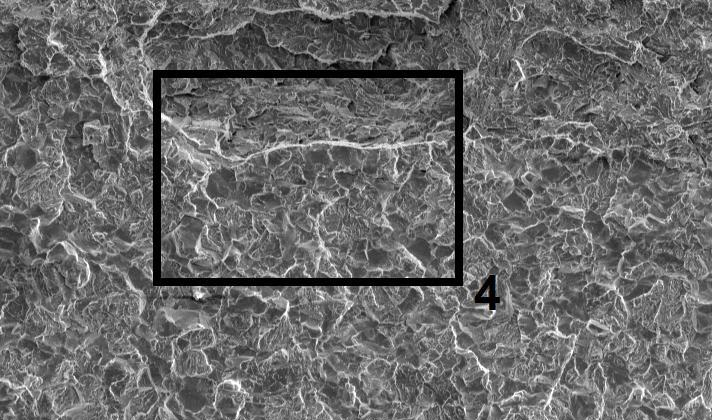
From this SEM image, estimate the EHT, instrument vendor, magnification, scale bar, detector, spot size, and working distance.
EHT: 20 kV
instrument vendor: FEI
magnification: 0.1 K X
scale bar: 200000 nm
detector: SE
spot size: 5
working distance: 29.6 mm